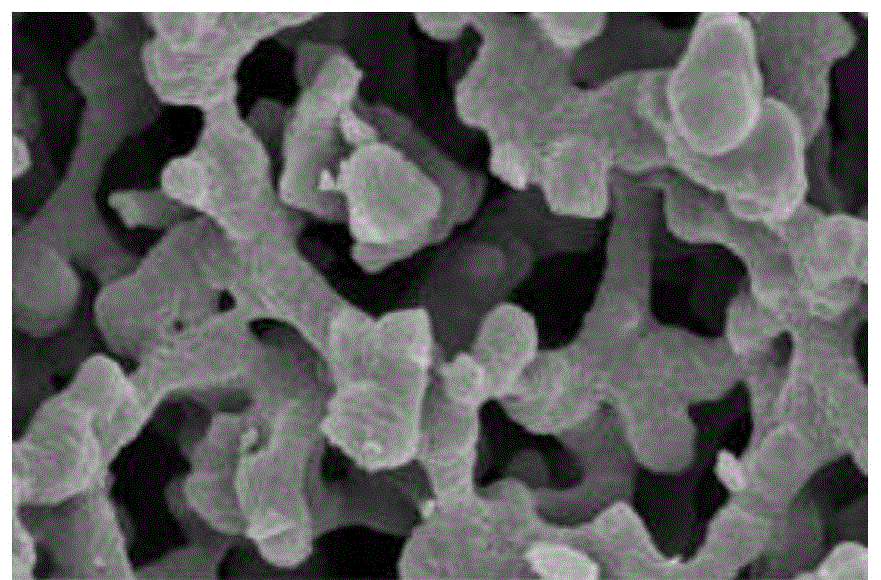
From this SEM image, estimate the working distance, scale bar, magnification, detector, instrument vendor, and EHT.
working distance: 5.4 mm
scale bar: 1000 nm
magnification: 50 K X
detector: TLD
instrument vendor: FEI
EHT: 5 kV